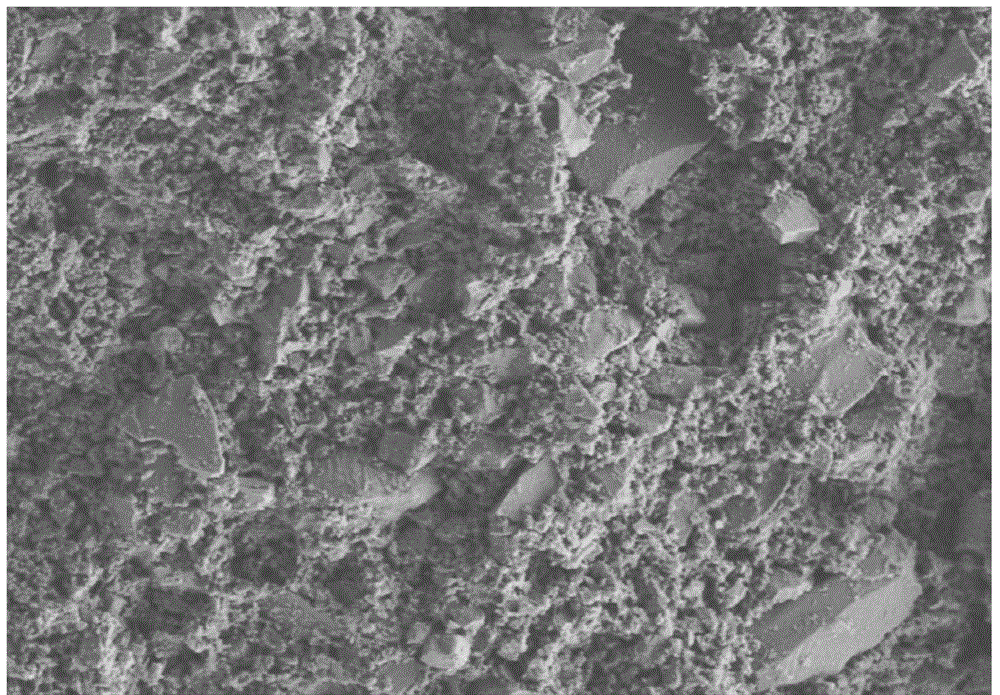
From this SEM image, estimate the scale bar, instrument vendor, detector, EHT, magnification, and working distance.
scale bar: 20000 nm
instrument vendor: Hitachi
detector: SE(L)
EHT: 5 kV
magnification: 2.5 K X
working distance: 8.9 mm